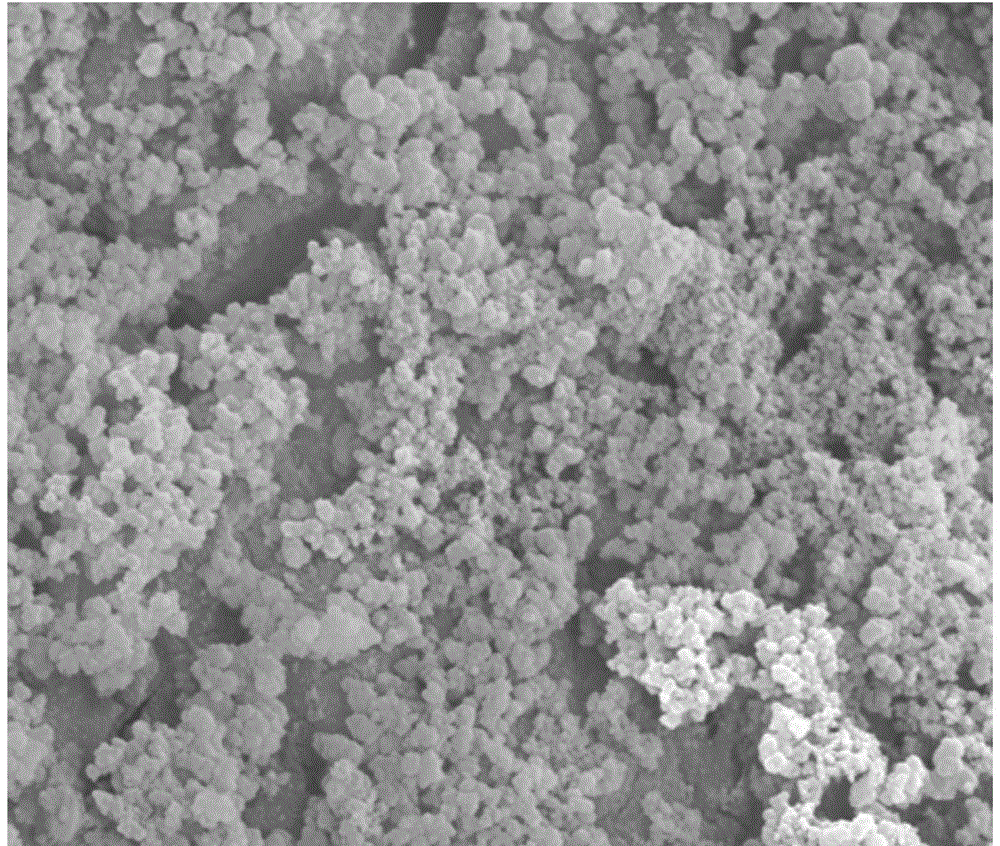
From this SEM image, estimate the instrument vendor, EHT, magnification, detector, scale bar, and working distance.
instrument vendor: FEI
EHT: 20 kV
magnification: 12 K X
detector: SE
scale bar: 5000 nm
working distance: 5.5 mm